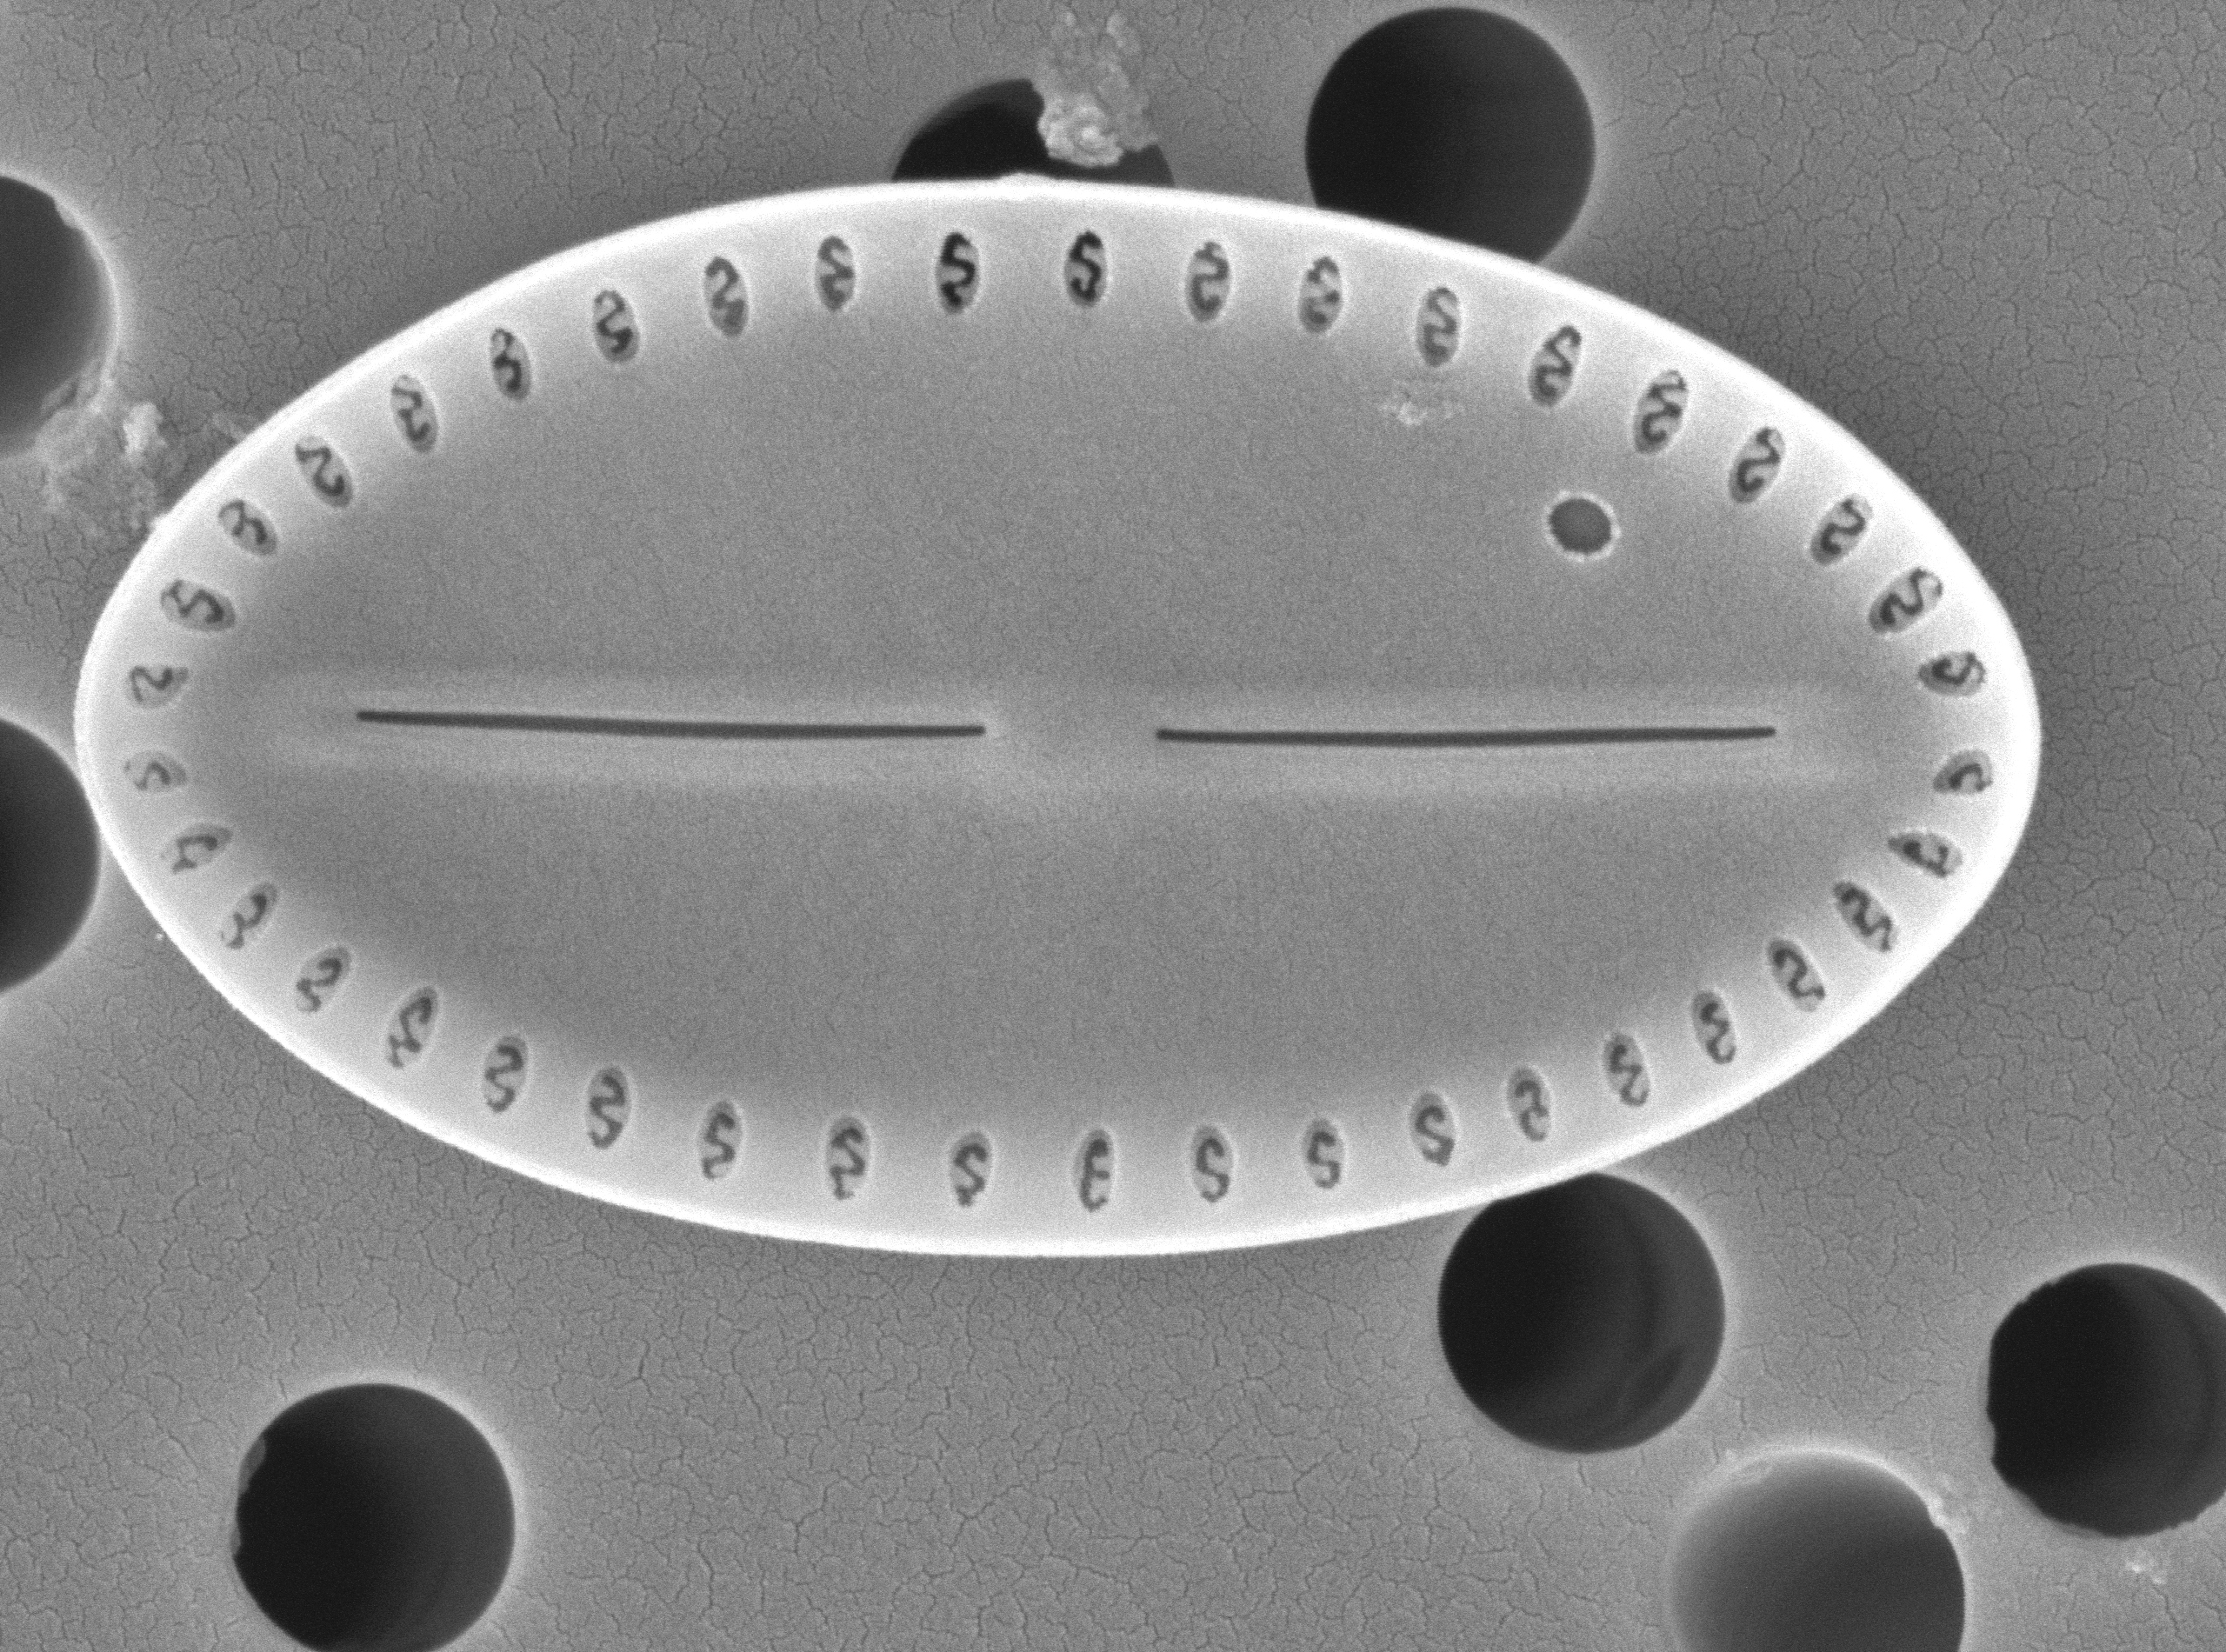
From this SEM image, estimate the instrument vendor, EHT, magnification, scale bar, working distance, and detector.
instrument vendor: JEOL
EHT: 5 kV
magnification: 20 K X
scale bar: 1000 nm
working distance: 9.4 mm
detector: SED